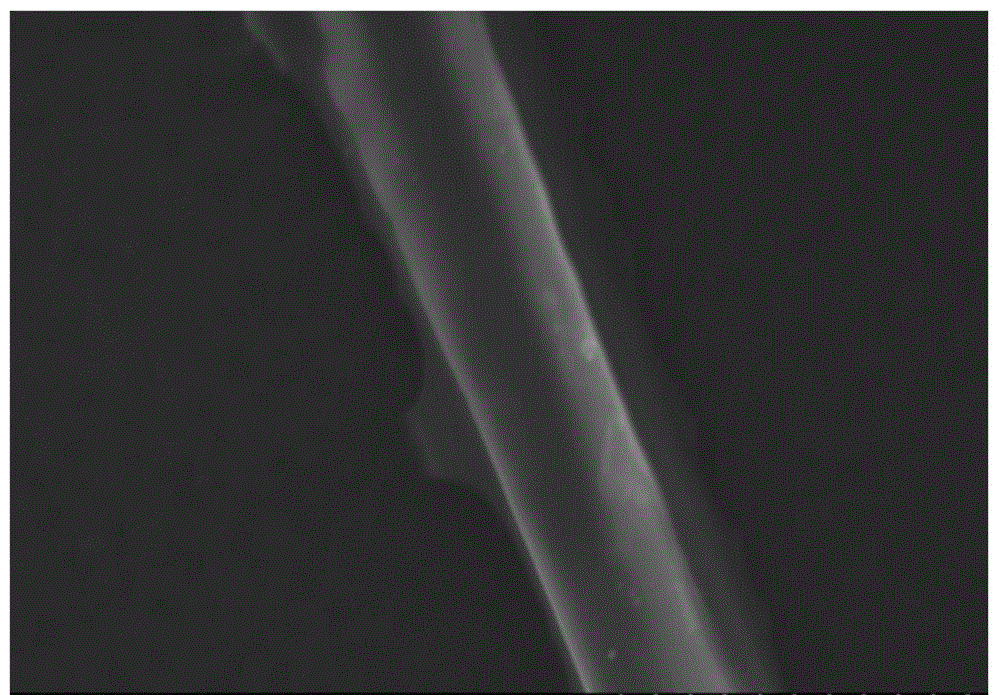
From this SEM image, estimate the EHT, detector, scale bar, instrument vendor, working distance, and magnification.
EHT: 15 kV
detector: SE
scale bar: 30000 nm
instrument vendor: Hitachi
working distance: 4.5 mm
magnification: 1.5 K X